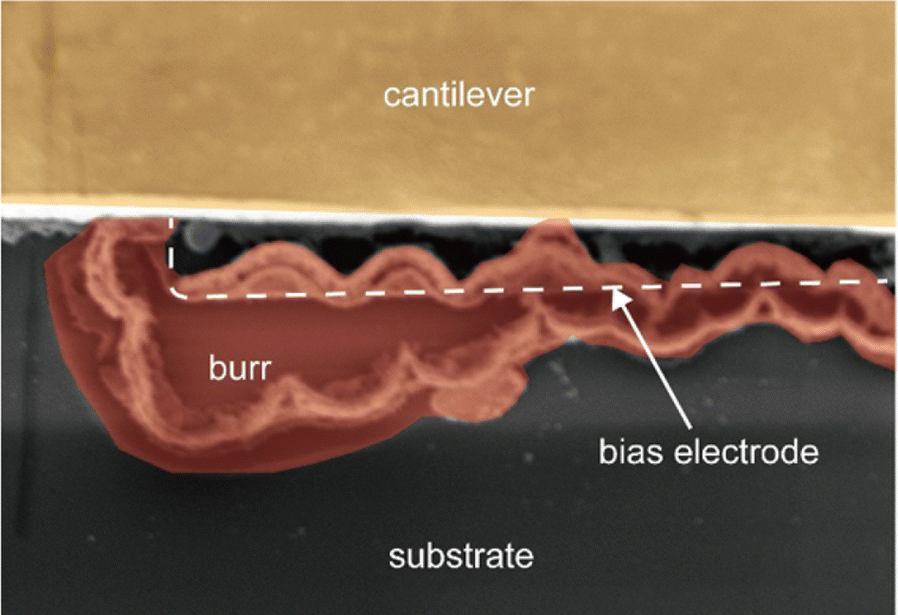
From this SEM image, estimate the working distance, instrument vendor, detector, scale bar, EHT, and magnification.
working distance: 4.9 mm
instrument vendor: Hitachi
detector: SE(M)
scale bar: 5000 nm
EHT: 1 kV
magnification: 7 K X